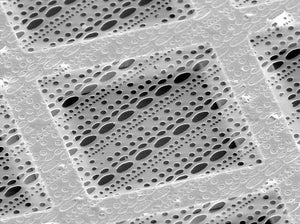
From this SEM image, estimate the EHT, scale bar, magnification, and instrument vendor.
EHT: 25 kV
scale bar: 30000 nm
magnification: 1 K X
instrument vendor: other